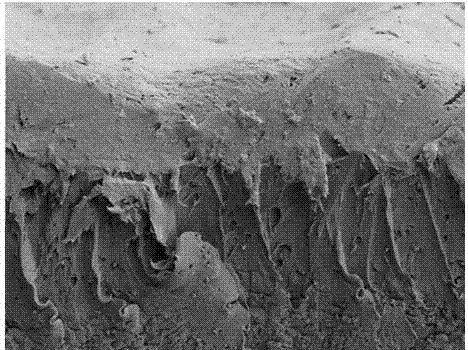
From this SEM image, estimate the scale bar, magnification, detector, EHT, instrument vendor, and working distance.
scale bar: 50000 nm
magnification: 1 K X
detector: SE(L)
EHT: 5 kV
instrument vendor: Hitachi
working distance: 10.3 mm